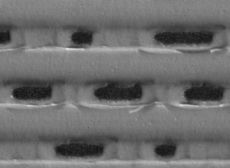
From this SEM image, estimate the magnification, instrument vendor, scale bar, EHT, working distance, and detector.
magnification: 20 K X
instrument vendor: JEOL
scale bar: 100 nm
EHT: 2 kV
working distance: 7.9 mm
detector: LEI